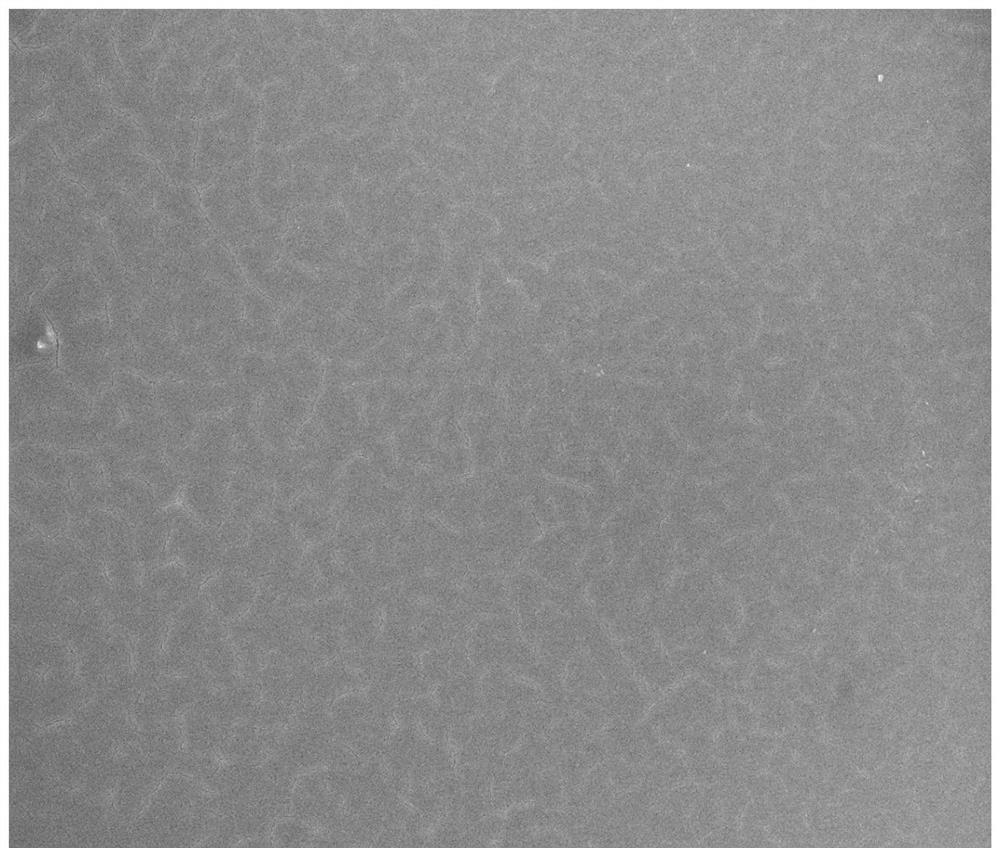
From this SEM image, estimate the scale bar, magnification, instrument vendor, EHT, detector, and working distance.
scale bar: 10000 nm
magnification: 5 K X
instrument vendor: FEI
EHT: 10 kV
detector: ETD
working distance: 6.5 mm